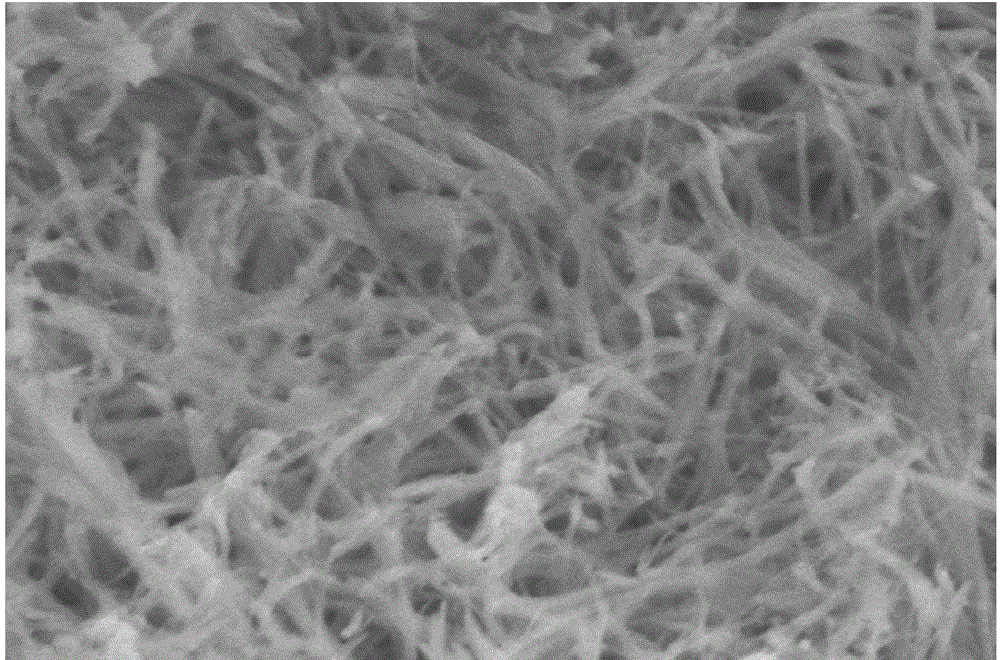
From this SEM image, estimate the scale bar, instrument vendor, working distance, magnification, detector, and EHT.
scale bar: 1000 nm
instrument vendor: Hitachi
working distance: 9.3 mm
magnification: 50 K X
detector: SE(M)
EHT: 5 kV